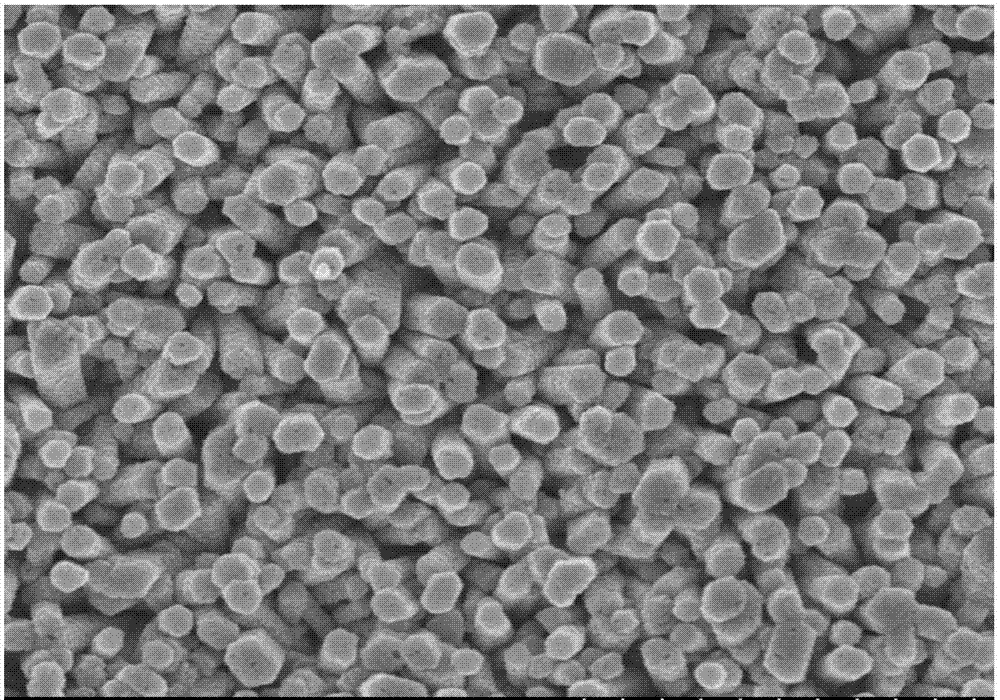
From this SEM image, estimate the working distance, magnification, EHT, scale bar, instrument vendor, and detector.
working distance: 9.6 mm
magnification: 25 K X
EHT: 5 kV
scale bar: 2000 nm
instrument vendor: Hitachi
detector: SE(U)